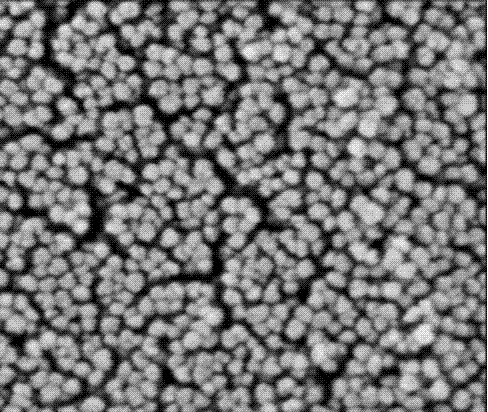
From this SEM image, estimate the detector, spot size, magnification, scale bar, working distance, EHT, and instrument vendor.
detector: TLD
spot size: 3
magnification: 100 K X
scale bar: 500 nm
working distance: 4.6 mm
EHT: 2 kV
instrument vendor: FEI